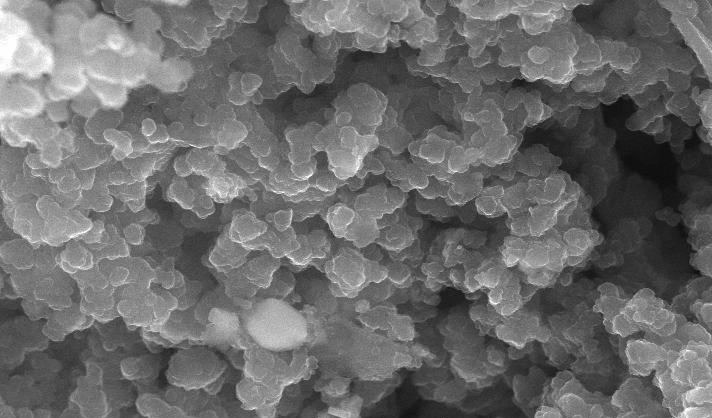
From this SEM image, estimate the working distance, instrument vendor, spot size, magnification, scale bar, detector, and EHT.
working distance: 4.4 mm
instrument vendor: FEI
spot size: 2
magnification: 20 K X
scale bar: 1000 nm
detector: TLD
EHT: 15 kV